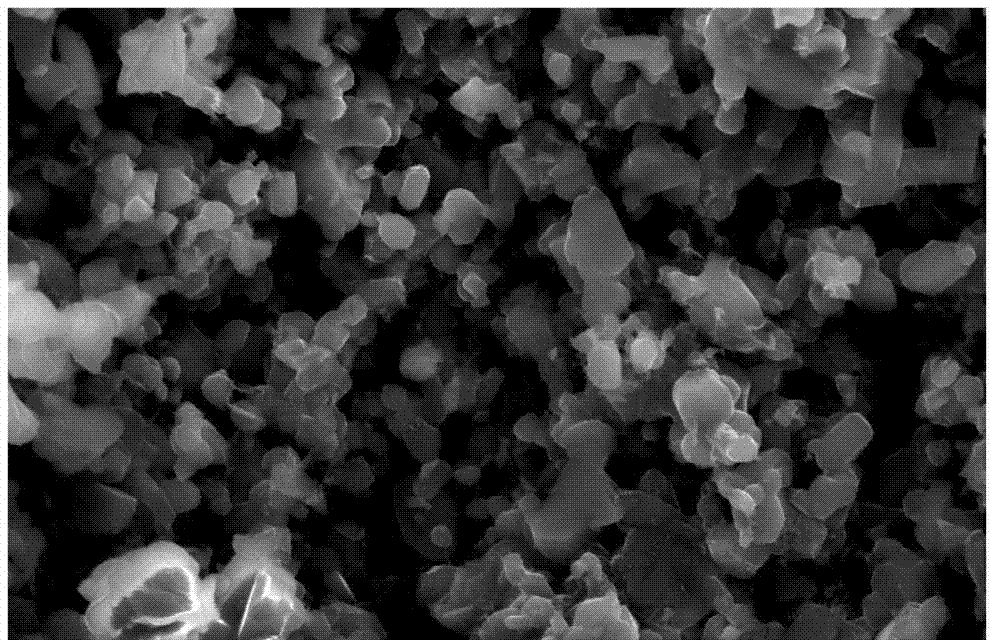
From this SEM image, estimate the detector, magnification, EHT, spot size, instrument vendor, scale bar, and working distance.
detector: SE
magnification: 5 K X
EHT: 25 kV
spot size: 4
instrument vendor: FEI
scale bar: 10000 nm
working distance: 7.9 mm